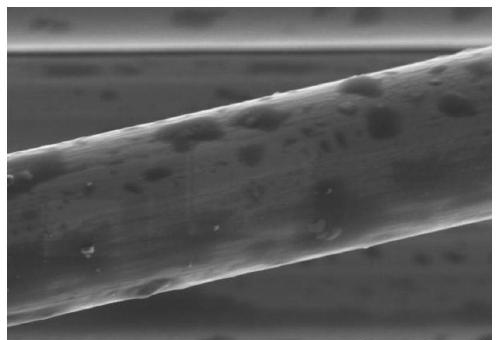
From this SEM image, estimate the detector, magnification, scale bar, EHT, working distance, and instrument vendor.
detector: SE(UL)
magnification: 6 K X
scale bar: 5000 nm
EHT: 3 kV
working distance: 8.6 mm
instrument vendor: Hitachi